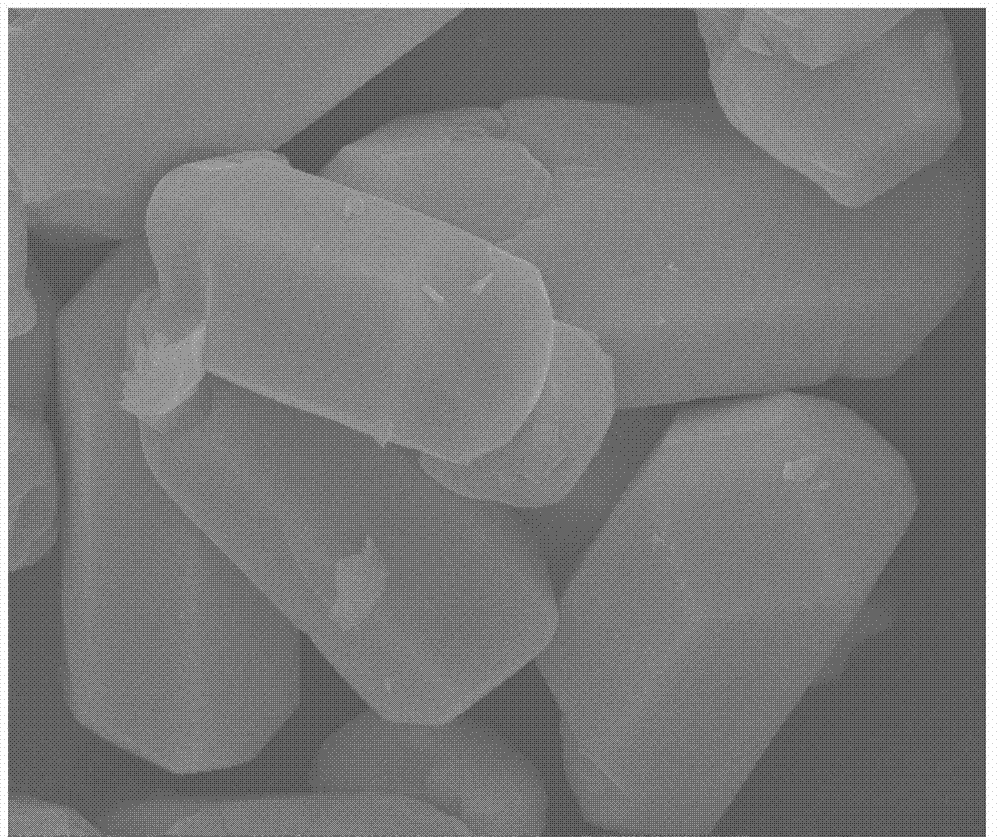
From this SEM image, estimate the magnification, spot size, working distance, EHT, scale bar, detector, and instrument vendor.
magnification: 20 K X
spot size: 3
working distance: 9.1 mm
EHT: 15 kV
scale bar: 5000 nm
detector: ETD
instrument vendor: FEI